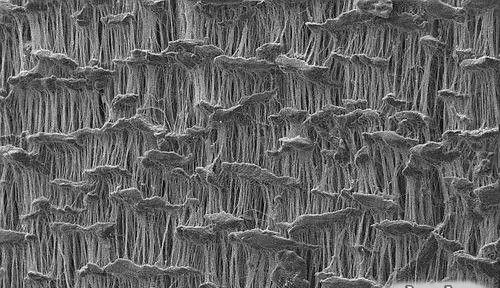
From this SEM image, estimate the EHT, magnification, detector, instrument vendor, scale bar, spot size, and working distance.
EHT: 10 kV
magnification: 1 K X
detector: SE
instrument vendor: FEI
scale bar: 50000 nm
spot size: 3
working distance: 10.5 mm